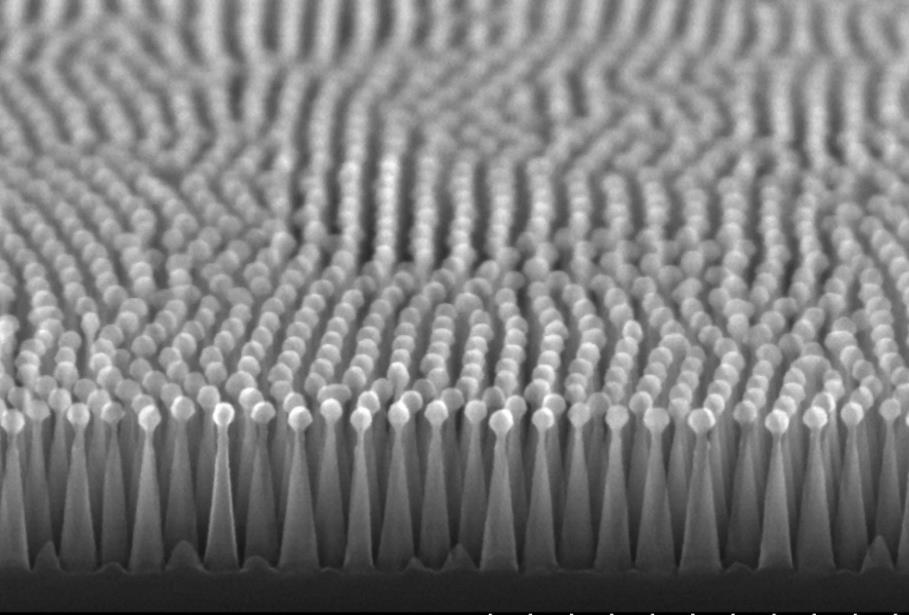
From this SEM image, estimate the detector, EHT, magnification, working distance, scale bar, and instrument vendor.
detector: SE(U,LA0)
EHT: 20 kV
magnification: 100 K X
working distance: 5.7 mm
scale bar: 500 nm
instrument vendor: Hitachi